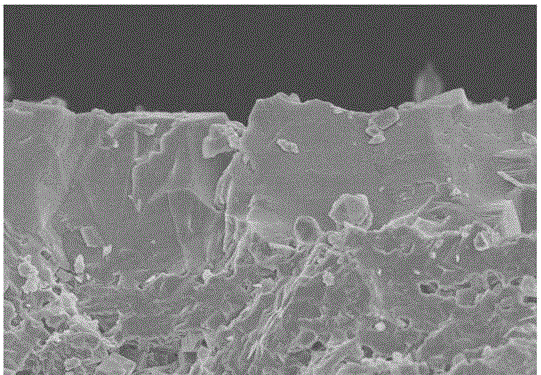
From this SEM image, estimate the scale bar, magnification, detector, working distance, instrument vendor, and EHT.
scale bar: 10000 nm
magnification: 4 K X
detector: SE(UL)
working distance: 9.6 mm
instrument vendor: Hitachi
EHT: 5 kV